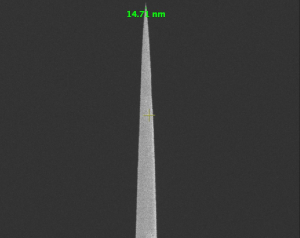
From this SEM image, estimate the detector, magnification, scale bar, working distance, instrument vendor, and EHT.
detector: TLD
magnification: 60 K X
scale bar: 2000 nm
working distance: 5.1 mm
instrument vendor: FEI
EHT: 3 kV